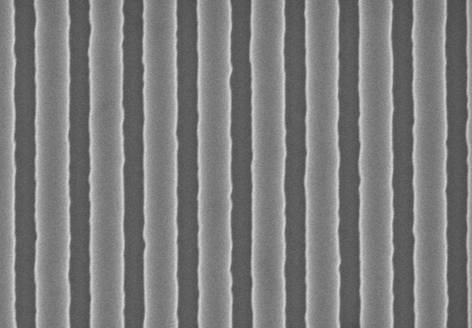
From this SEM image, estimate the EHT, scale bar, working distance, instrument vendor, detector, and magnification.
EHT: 3 kV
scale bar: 1000 nm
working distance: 8.4 mm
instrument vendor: Hitachi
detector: SE(U)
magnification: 50 K X